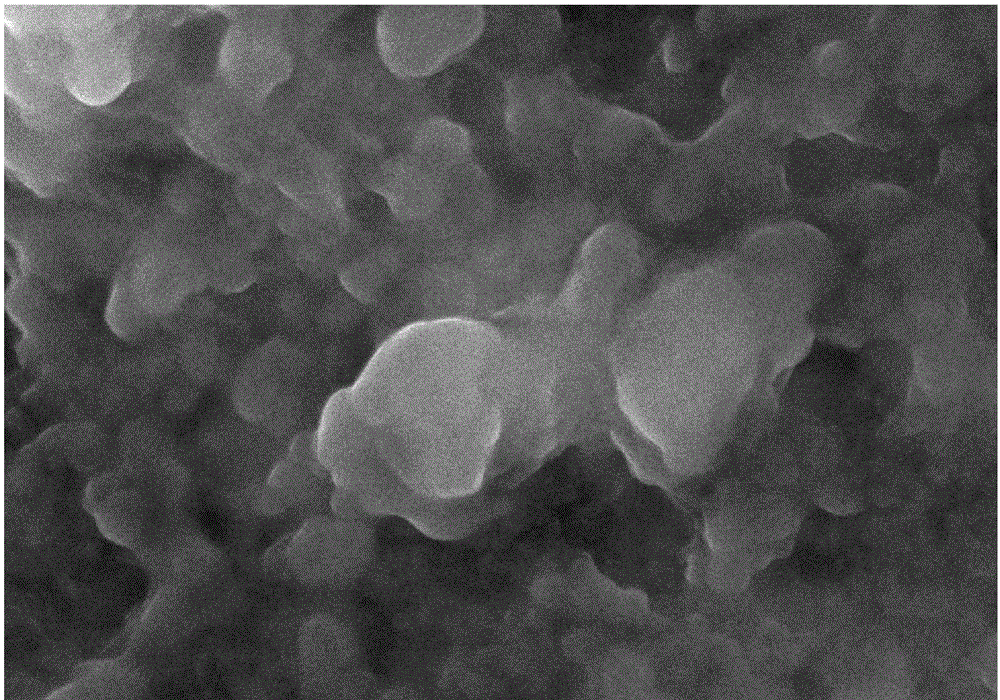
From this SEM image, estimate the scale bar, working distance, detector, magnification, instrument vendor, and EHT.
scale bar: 200 nm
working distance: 7.5 mm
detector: InlensDuo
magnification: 50 K X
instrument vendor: Zeiss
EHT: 10 kV